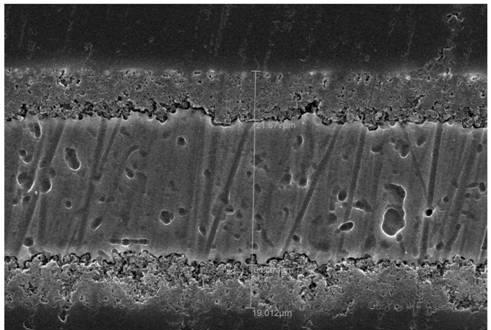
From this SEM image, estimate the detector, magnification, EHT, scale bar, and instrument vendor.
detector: SEI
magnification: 0.6 K X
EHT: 30 kV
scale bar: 20000 nm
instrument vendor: JEOL